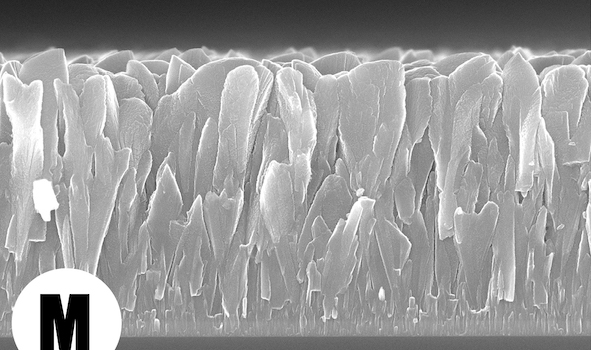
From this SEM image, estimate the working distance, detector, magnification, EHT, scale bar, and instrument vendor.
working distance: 5 mm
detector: SEI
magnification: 30 K X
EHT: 15 kV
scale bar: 100 nm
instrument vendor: JEOL